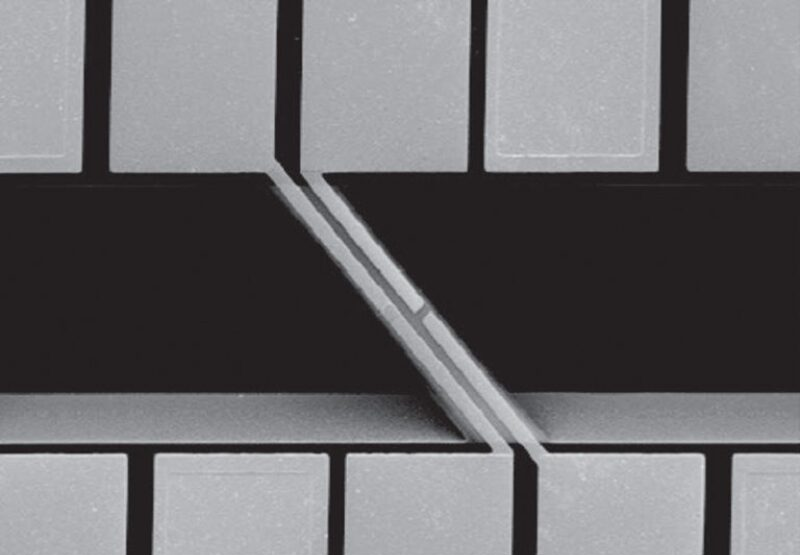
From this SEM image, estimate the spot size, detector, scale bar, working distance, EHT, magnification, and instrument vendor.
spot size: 3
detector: SE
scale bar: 50000 nm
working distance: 7.1 mm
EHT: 10 kV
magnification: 1.2 K X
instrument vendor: FEI